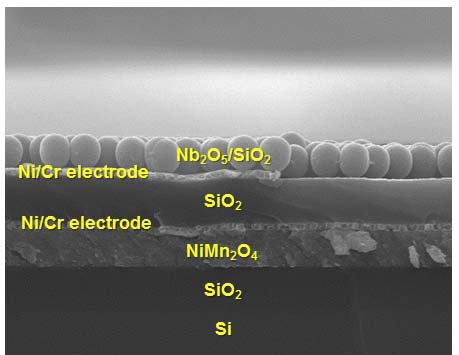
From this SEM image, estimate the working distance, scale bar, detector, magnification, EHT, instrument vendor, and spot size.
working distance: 9.6 mm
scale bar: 2000 nm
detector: SE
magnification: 10 K X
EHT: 15 kV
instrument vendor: FEI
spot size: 3